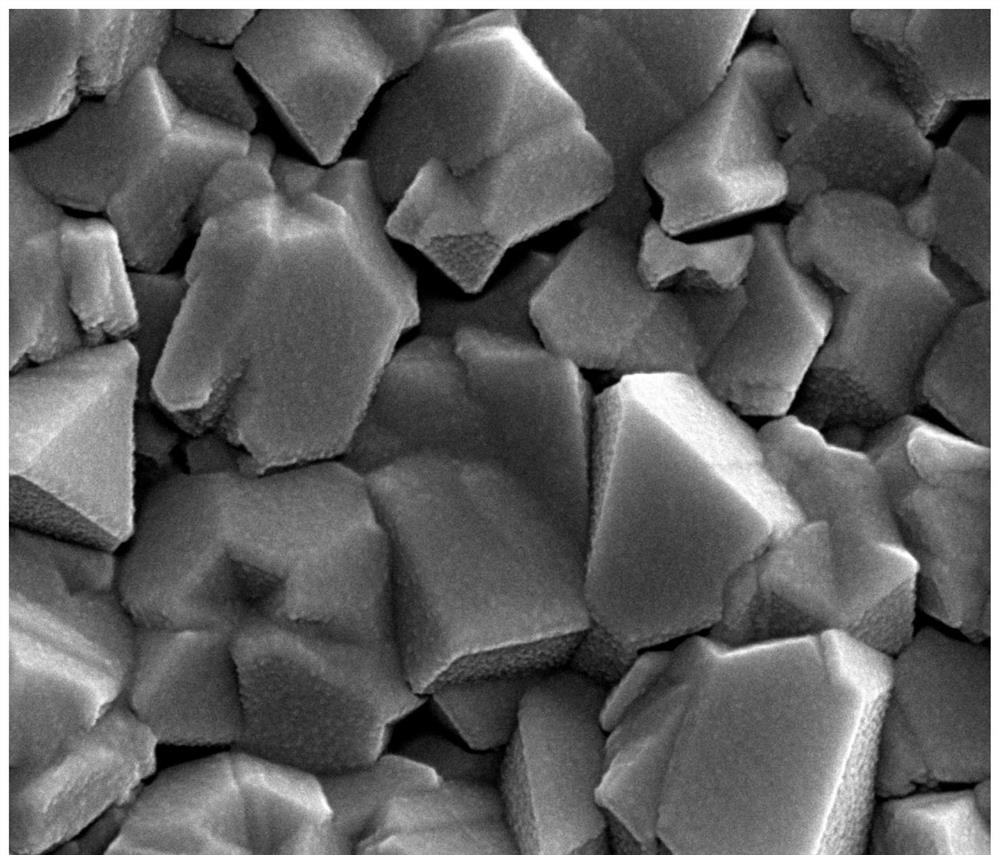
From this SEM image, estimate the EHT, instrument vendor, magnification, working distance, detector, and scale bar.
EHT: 20 kV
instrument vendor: FEI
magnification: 80 K X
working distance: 5.2 mm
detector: SE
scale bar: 1000 nm